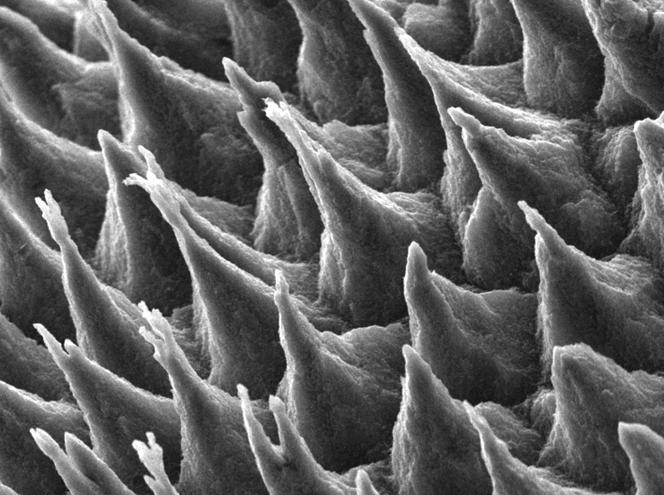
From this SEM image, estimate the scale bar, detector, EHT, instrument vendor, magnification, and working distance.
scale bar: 1000 nm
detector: LED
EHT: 5 kV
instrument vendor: JEOL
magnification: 5 K X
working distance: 10.2 mm